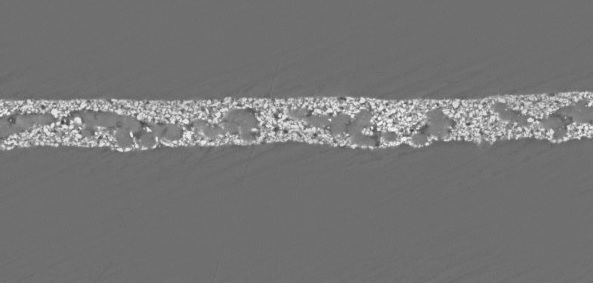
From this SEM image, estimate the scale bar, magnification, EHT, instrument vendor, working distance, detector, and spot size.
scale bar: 50000 nm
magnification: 0.5 K X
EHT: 20 kV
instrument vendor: FEI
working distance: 10.5 mm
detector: BSE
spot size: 6